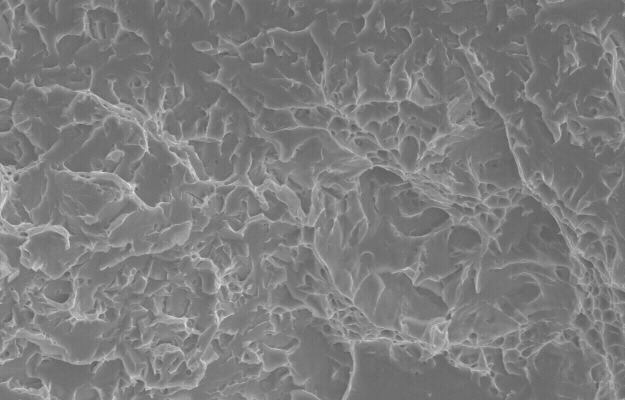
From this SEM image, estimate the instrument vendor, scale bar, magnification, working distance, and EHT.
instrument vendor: JEOL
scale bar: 10000 nm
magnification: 1.5 K X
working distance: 35 mm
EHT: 20 kV